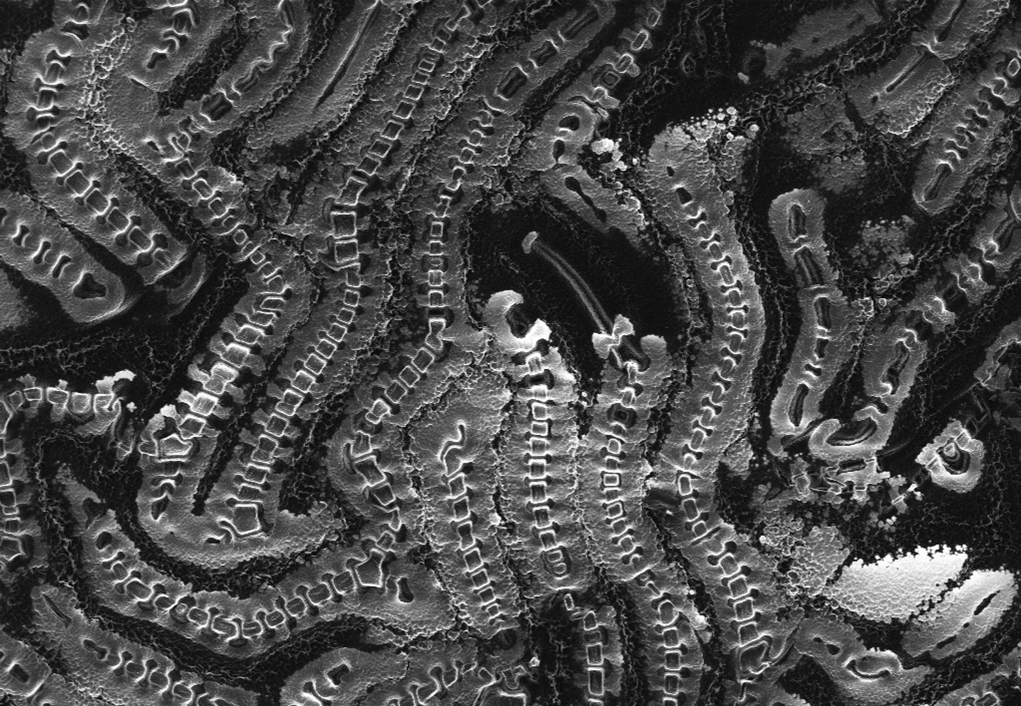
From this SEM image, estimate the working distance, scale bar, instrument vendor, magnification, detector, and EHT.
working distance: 5 mm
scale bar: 20000 nm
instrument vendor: Zeiss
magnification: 0.606 K X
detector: SE2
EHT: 3 kV